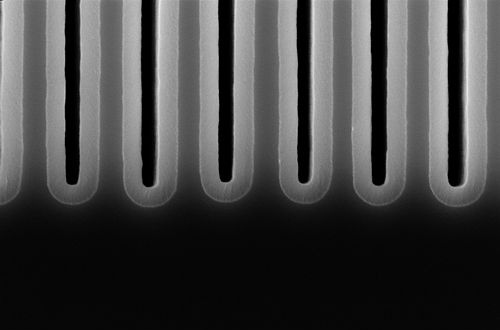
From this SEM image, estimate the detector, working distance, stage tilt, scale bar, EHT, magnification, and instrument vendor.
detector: InLens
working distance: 3.4 mm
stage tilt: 0°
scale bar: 200 nm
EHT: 5 kV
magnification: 115.72 K X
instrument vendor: Zeiss